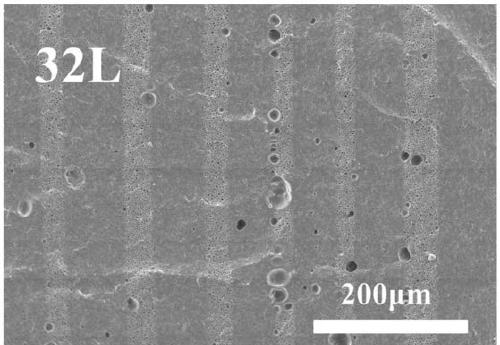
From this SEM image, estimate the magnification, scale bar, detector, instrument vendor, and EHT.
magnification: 0.2 K X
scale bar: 200000 nm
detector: SED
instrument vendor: other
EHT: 10 kV